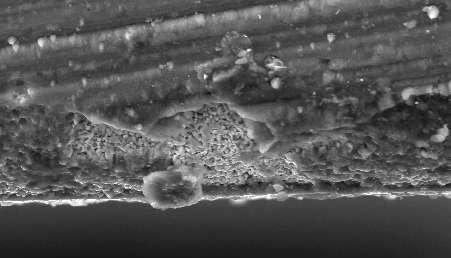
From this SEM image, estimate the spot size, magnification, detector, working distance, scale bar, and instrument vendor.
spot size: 4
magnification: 1.5 K X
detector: SE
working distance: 10.6 mm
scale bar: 20000 nm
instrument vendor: FEI